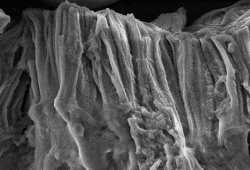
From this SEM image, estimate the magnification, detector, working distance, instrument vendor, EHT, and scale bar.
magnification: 20 K X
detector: SE(M)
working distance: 20.2 mm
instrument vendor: Hitachi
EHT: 3 kV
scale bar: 2000 nm